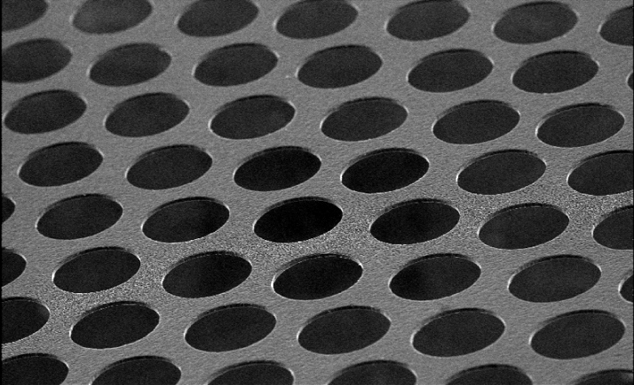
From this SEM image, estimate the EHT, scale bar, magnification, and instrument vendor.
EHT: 25 kV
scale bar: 500000 nm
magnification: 0.05 K X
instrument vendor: JEOL